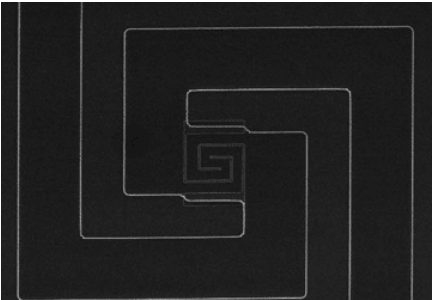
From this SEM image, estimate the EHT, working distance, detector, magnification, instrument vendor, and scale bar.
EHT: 5 kV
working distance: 13.7 mm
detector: SE(M)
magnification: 1.5 K X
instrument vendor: Hitachi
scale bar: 30000 nm